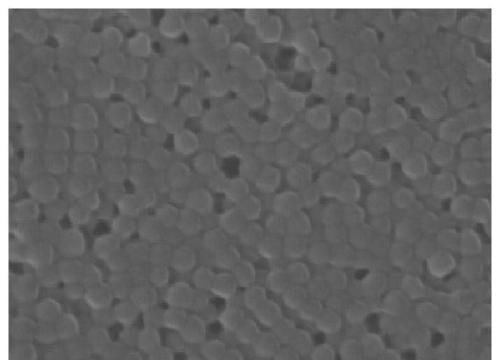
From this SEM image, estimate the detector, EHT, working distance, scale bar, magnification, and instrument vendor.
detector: SEI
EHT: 10 kV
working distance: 6.9 mm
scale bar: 100 nm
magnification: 100 K X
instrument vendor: JEOL